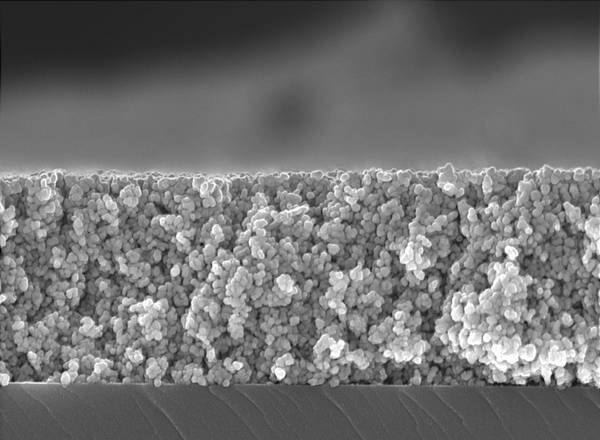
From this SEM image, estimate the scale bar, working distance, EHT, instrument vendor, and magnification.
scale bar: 500 nm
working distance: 3 mm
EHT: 4 kV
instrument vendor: Zeiss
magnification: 50 K X